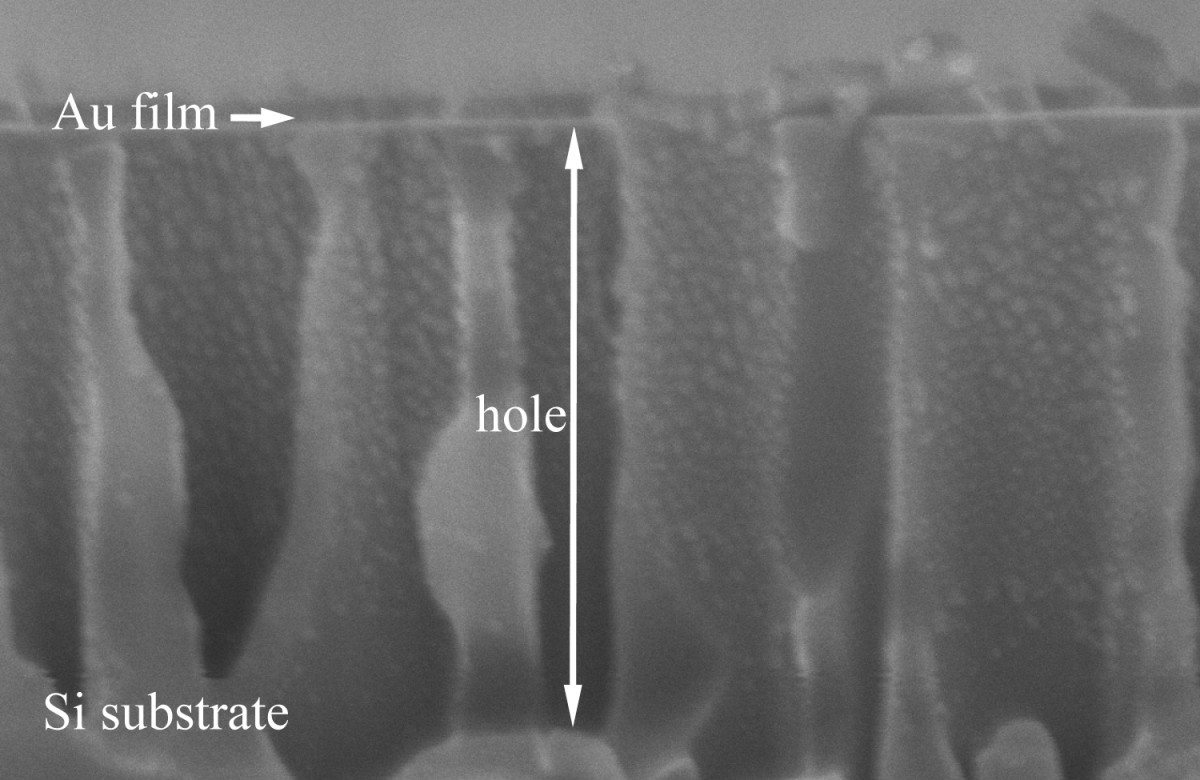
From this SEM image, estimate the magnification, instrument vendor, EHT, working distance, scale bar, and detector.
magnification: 150 K X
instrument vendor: Hitachi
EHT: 5 kV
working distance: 8.8 mm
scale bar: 300 nm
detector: SE(M)